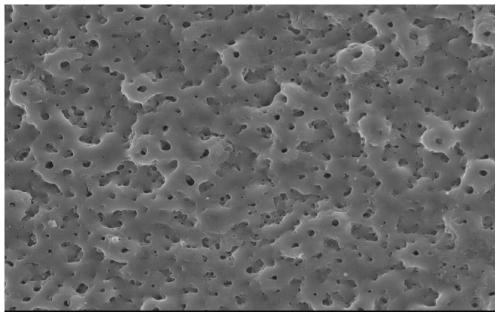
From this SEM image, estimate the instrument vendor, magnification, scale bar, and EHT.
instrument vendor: other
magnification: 1 K X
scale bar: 10000 nm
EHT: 30 kV